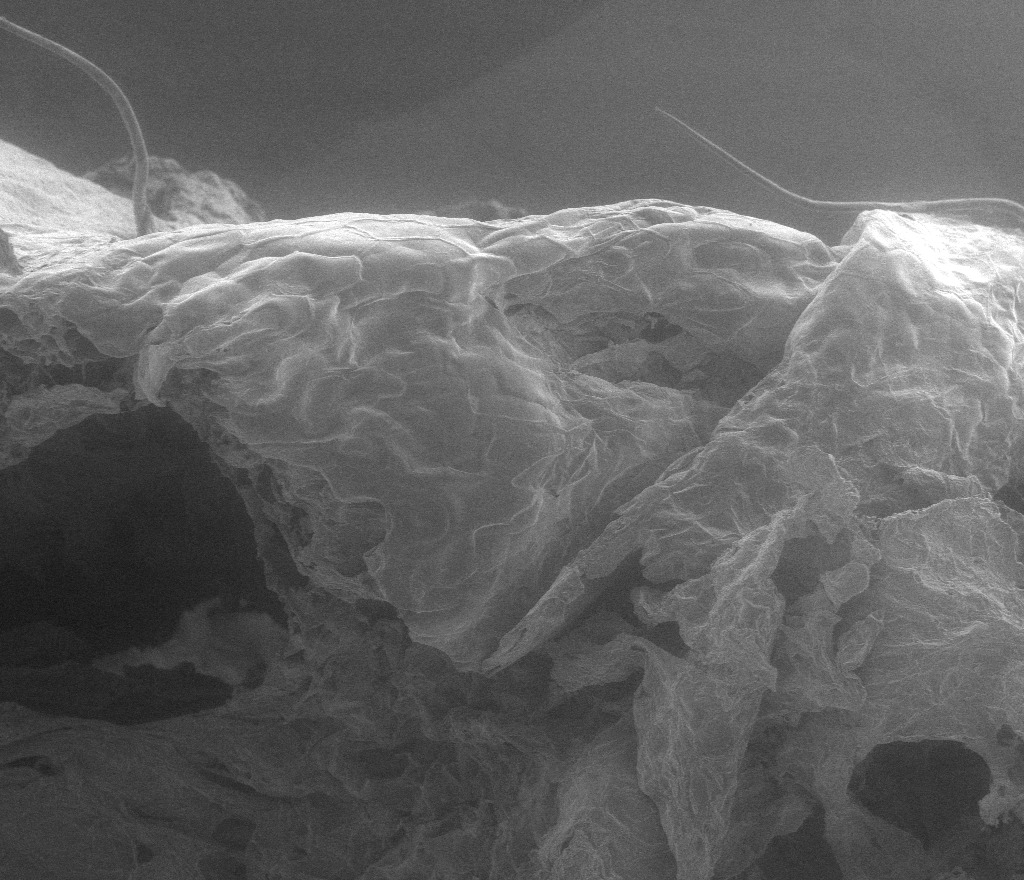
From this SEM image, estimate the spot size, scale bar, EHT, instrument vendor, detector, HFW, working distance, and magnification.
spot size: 3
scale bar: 500000 nm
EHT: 5 kV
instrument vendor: FEI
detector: LFD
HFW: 1860 µm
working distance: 15.7 mm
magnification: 0.16 K X